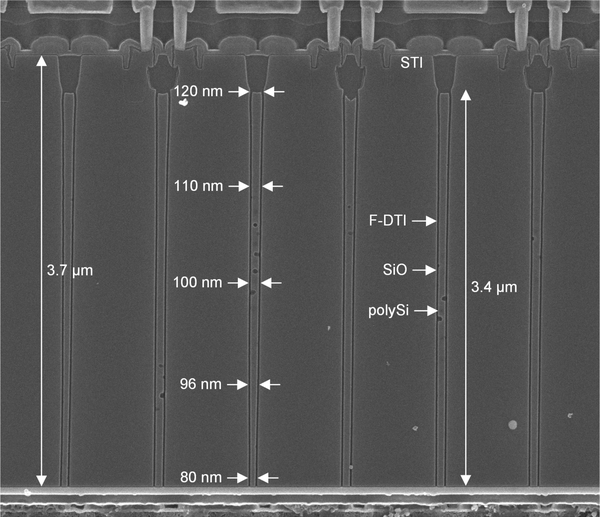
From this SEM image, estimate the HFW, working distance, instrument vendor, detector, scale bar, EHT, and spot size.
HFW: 5.08 µm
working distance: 5.2 mm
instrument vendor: FEI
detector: TLD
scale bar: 1000 nm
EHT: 5 kV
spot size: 2.5